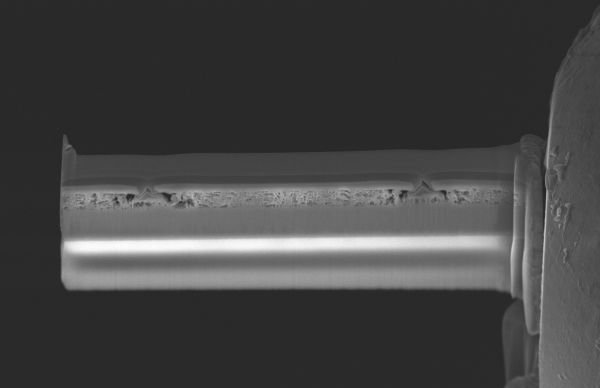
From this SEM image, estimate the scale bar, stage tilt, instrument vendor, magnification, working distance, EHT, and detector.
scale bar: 5000 nm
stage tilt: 59°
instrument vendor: FEI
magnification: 8 K X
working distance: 7 mm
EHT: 10 kV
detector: T2